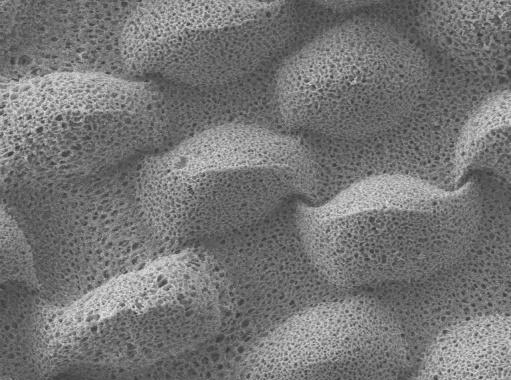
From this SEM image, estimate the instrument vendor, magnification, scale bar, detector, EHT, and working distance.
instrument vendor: JEOL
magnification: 0.8 K X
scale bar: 10000 nm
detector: LEI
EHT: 10 kV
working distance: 8 mm